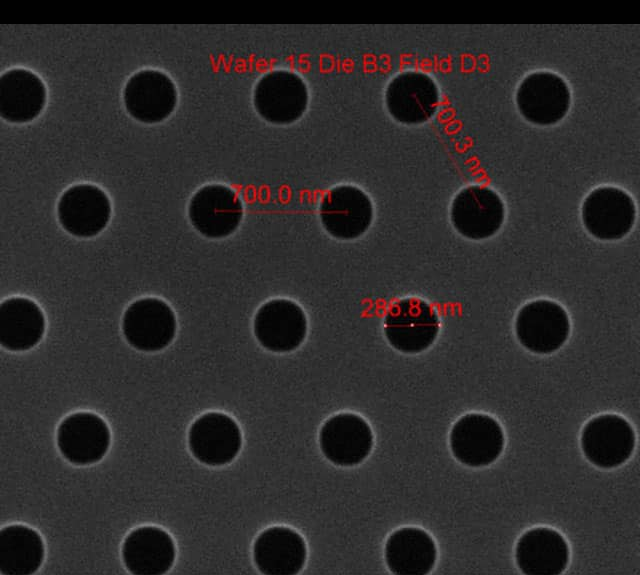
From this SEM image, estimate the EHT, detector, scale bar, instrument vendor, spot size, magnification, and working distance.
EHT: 10 kV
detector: ETD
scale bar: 1000 nm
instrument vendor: FEI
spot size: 1.5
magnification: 75 K X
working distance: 10.2 mm